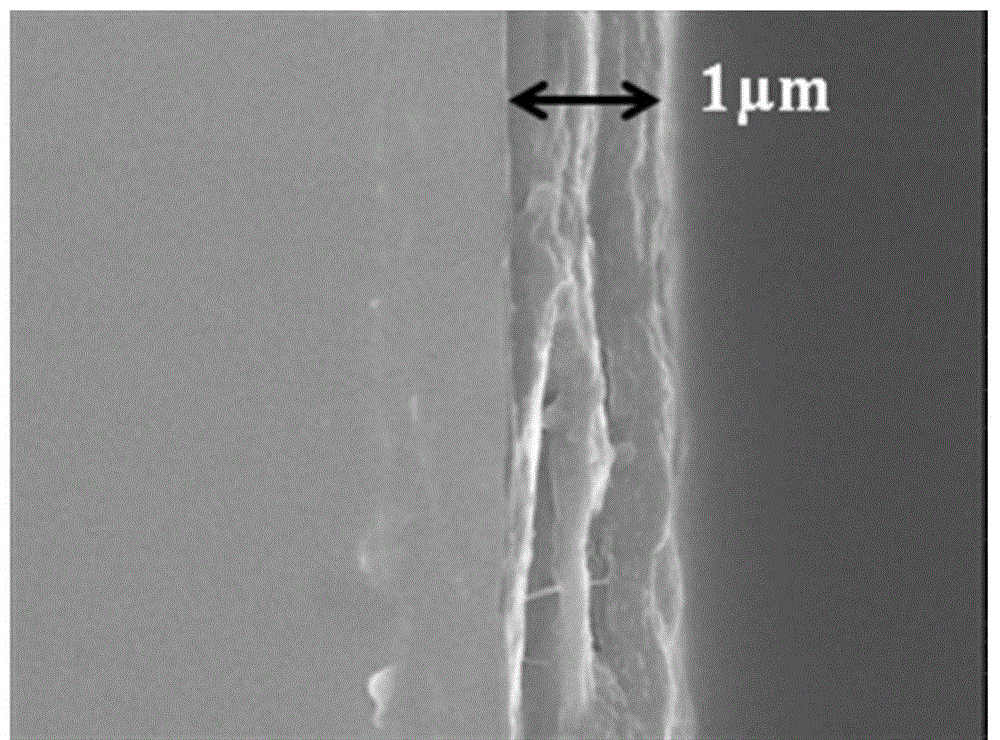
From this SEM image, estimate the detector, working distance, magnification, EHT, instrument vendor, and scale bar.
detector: SEI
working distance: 6 mm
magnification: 10 K X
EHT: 10 kV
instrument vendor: JEOL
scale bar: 1000 nm